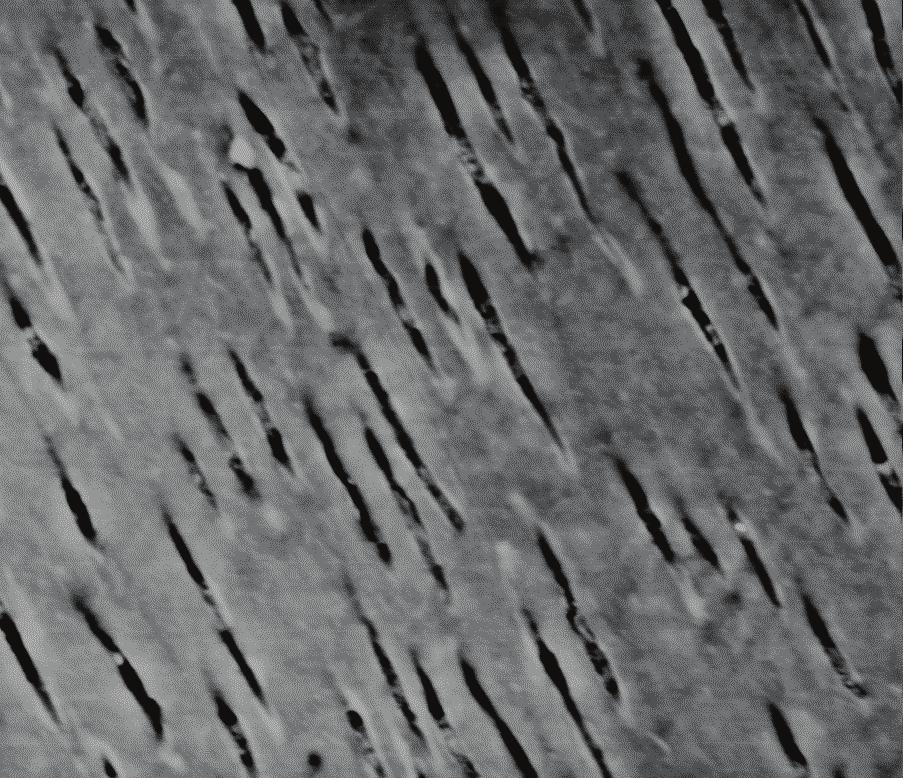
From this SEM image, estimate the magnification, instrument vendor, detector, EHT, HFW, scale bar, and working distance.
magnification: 1.183 K X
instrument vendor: FEI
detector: SSD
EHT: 25 kV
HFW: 110 µm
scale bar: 20000 nm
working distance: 9.9 mm